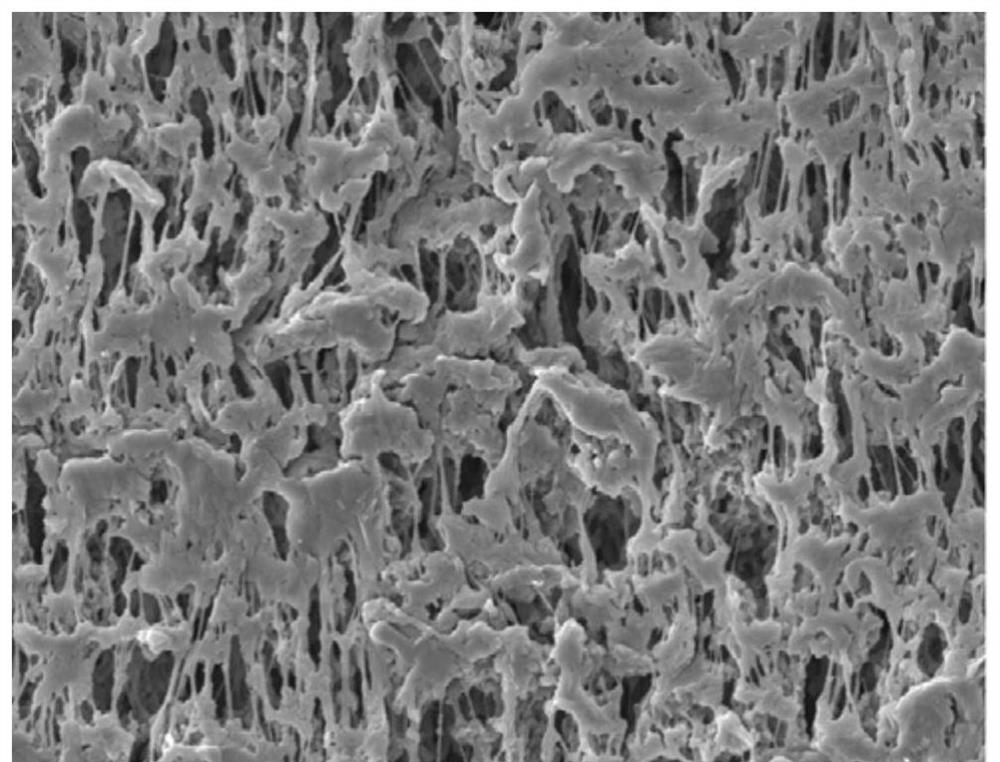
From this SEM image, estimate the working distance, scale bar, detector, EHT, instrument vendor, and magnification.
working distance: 7.3 mm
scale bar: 1000 nm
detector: LED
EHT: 3 kV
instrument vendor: JEOL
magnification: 10 K X